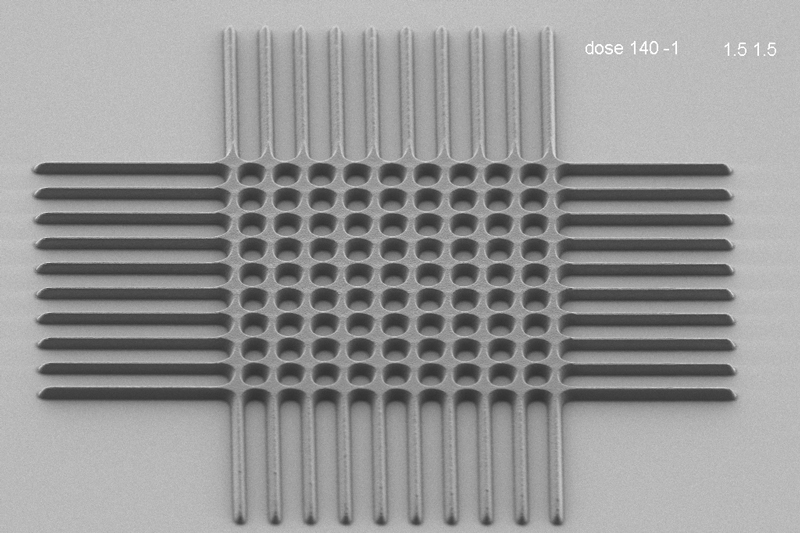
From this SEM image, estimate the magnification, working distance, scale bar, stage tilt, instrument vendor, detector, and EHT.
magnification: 1.73 K X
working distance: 6.7 mm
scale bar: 10000 nm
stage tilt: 45°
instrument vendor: Zeiss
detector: HE-SE2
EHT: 1 kV